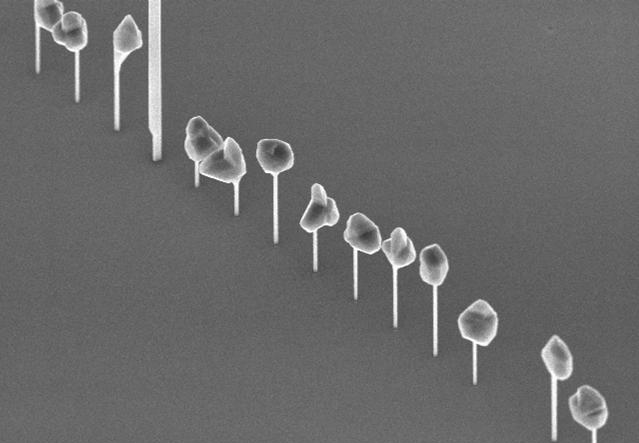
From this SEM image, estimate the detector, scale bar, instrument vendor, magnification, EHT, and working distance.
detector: SE(U)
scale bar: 3000 nm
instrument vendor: Hitachi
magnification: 15 K X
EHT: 5 kV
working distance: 5.5 mm